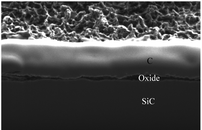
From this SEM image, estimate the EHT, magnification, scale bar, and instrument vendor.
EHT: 30 kV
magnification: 10 K X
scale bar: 1000 nm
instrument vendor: JEOL